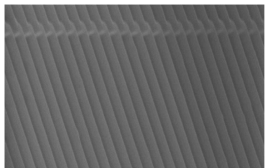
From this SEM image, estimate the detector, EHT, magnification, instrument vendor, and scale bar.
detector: SE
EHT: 20 kV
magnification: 7 K X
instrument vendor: Hitachi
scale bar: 4290 nm